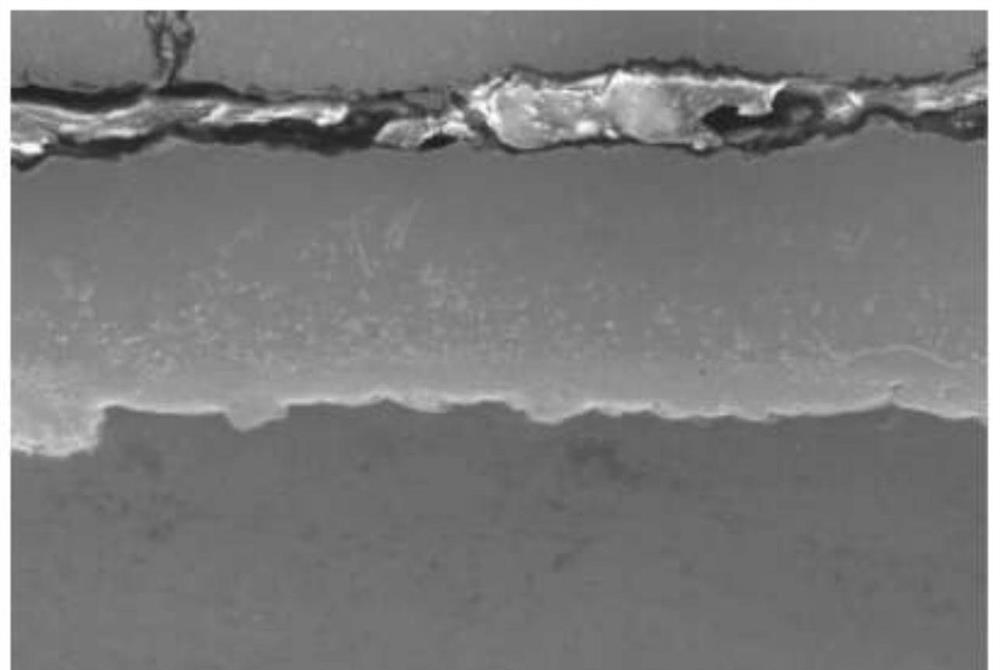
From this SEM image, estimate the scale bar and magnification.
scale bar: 50000 nm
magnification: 0.5 K X